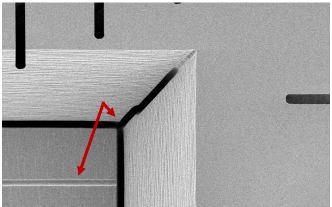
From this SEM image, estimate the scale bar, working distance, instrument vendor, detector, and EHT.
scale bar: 100000 nm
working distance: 9.7 mm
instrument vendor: Zeiss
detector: SE2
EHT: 2 kV